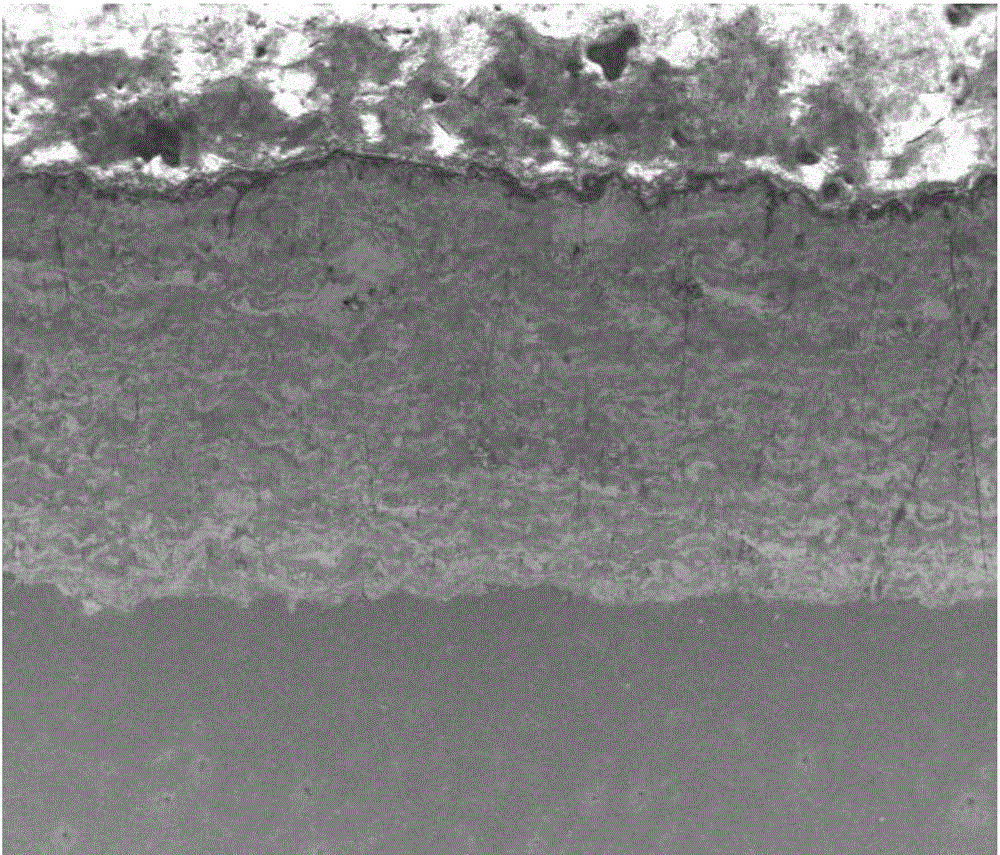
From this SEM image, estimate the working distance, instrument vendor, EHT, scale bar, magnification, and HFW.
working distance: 5.4 mm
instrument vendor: FEI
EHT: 7 kV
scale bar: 500000 nm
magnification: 0.2 K X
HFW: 1490 µm